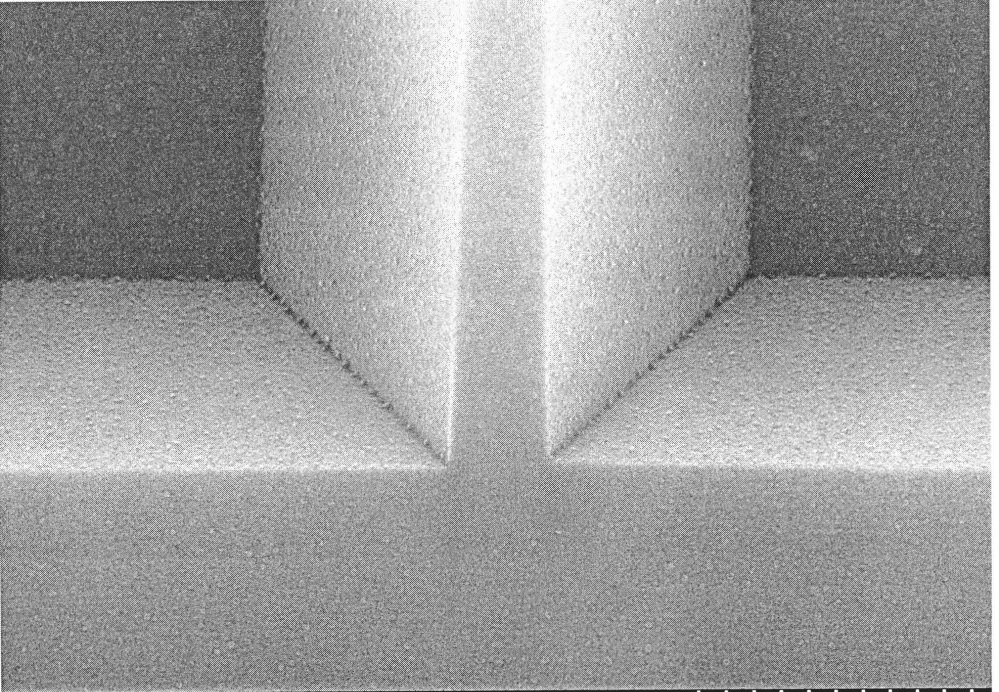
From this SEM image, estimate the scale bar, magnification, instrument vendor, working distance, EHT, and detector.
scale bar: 500 nm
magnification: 70 K X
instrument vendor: Hitachi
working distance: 4.9 mm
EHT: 15 kV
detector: SE(U)